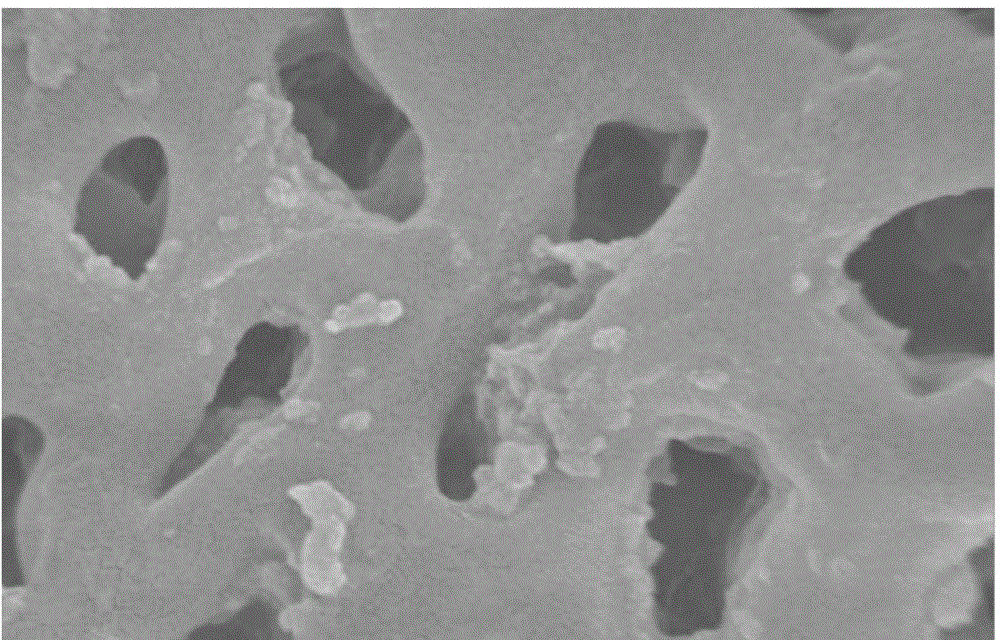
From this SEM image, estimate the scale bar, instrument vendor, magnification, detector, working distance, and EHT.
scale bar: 1000 nm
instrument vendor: Hitachi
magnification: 50 K X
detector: SE(M)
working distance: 9.1 mm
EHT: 5 kV